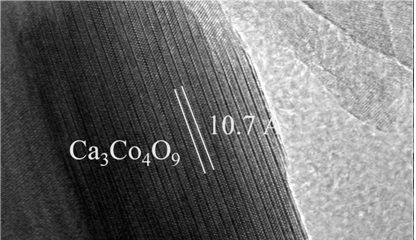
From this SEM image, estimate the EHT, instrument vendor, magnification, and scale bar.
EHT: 200 kV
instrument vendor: FEI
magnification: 1050 K X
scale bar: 10 nm